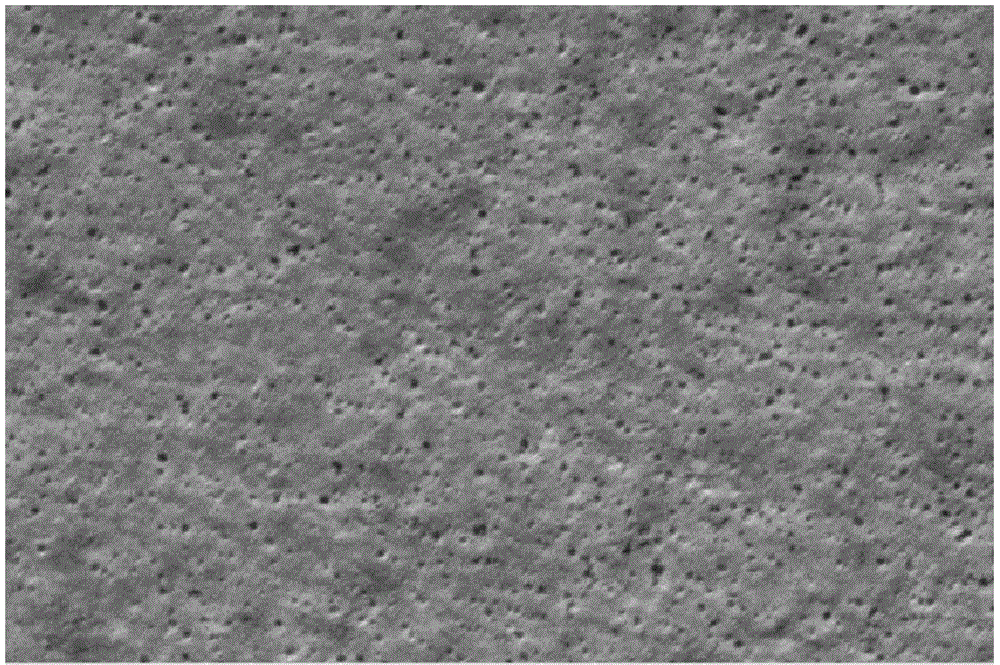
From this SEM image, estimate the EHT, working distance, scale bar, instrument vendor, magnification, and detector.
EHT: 5 kV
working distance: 9.9 mm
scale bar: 2000 nm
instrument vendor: FEI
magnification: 60 K X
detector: ETD